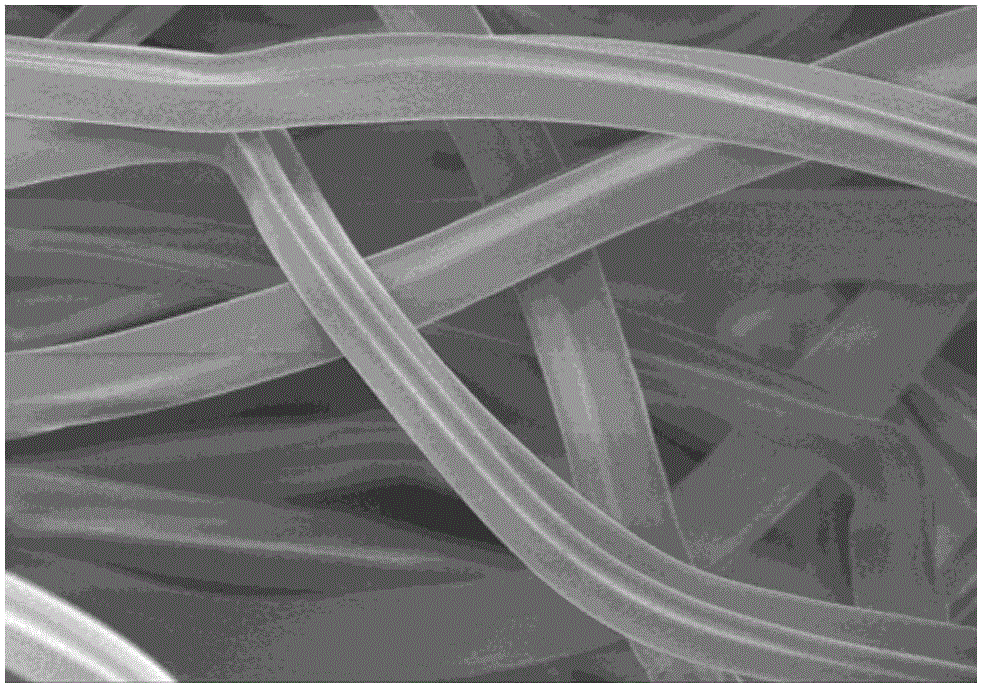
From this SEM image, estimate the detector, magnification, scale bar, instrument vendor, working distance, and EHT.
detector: SE(M)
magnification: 0.5 K X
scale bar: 100000 nm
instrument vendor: Hitachi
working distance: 8.7 mm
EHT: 3 kV